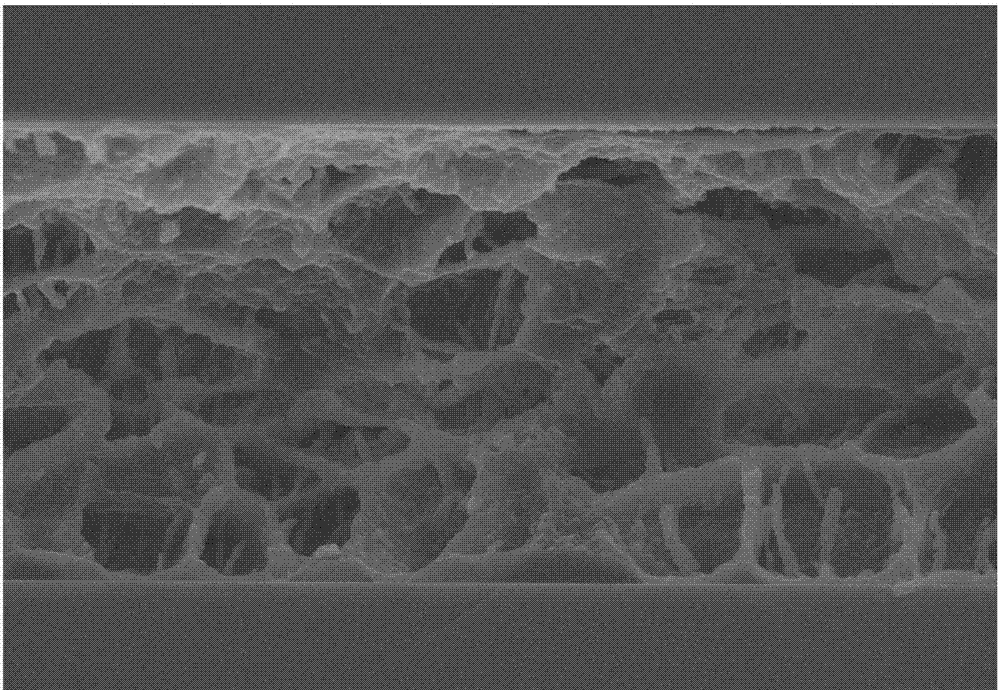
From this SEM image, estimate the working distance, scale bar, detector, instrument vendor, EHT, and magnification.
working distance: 6.4 mm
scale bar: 10000 nm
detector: SE(M)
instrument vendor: Hitachi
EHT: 10 kV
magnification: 4.5 K X